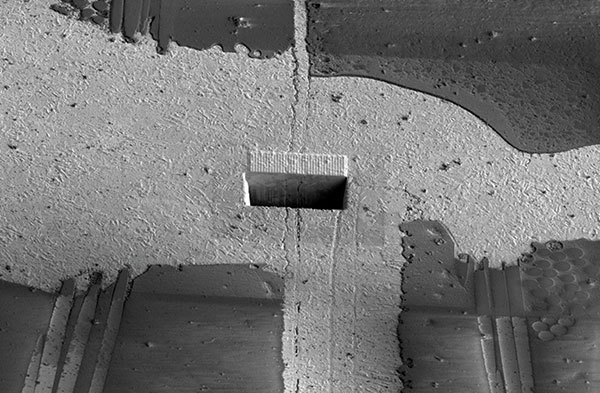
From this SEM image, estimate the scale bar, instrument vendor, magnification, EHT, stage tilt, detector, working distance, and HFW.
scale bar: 50000 nm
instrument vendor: FEI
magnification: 1 K X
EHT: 2 kV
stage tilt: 52°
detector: SE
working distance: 4 mm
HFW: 207 µm